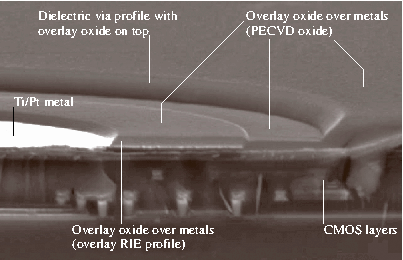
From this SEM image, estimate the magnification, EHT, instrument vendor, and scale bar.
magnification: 4 K X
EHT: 20 kV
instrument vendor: JEOL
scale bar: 50000 nm